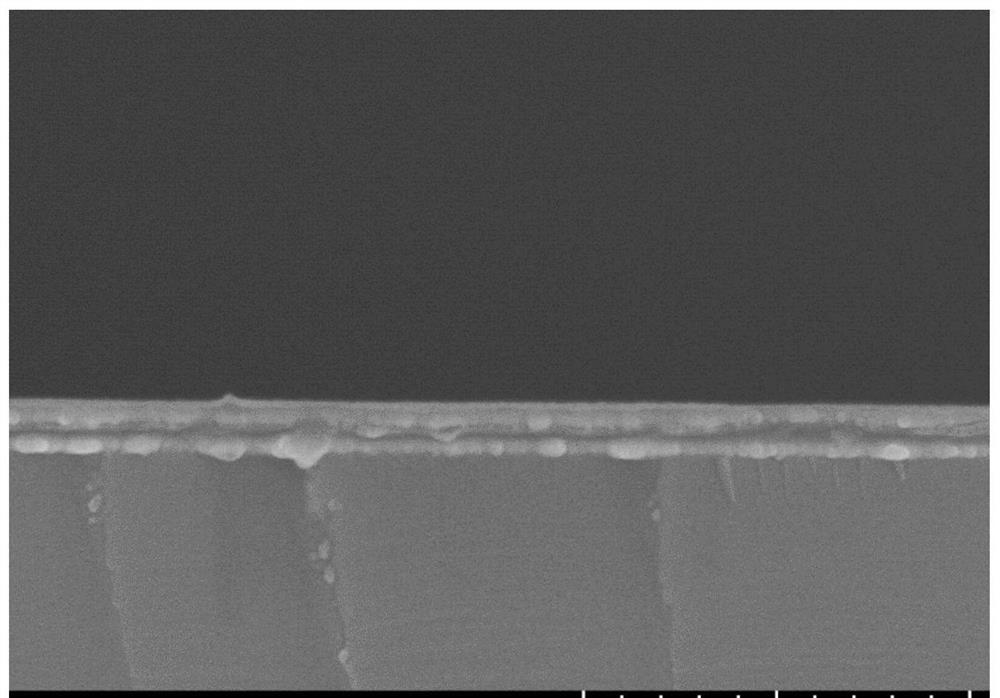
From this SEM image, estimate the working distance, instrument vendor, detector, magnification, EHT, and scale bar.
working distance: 10.7 mm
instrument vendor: Hitachi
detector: LA1(U)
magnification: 50 K X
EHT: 5 kV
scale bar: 1000 nm